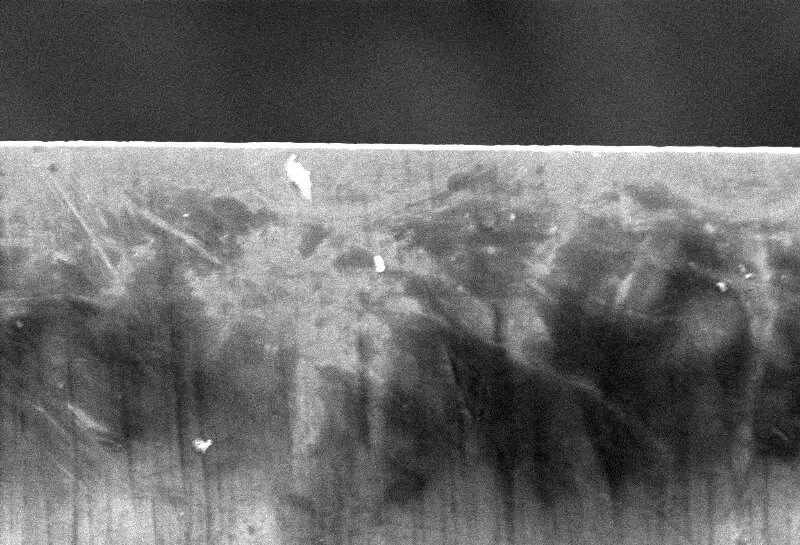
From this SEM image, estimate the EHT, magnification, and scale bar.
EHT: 15 kV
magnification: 0.6 K X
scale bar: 50000 nm